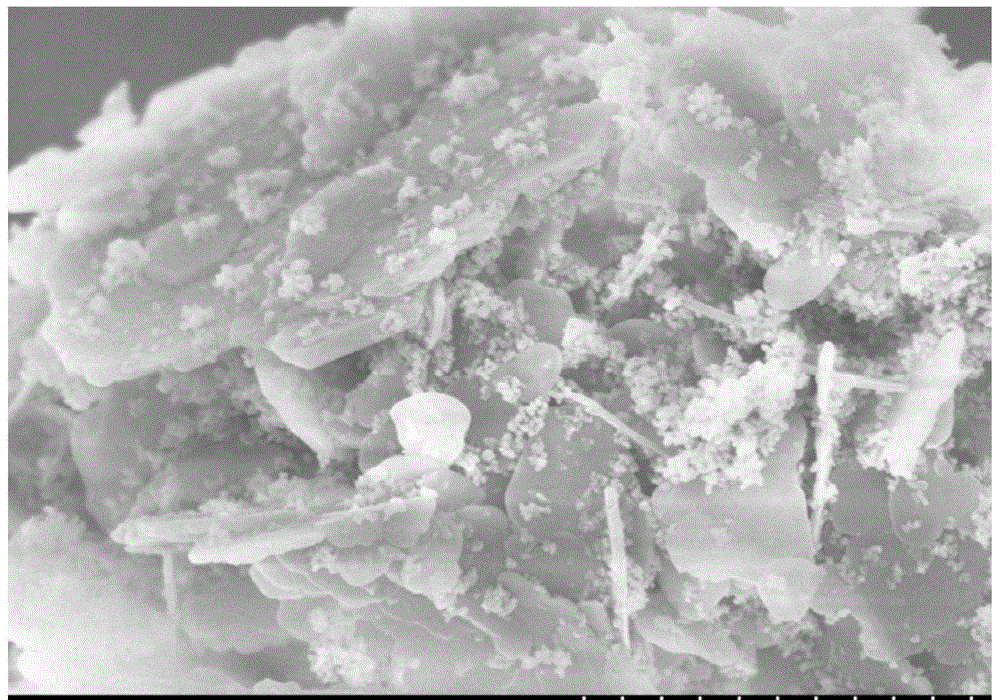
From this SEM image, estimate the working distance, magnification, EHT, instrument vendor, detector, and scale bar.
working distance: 8.4 mm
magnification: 25 K X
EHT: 5 kV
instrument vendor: Hitachi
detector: SE(M)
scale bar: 2000 nm